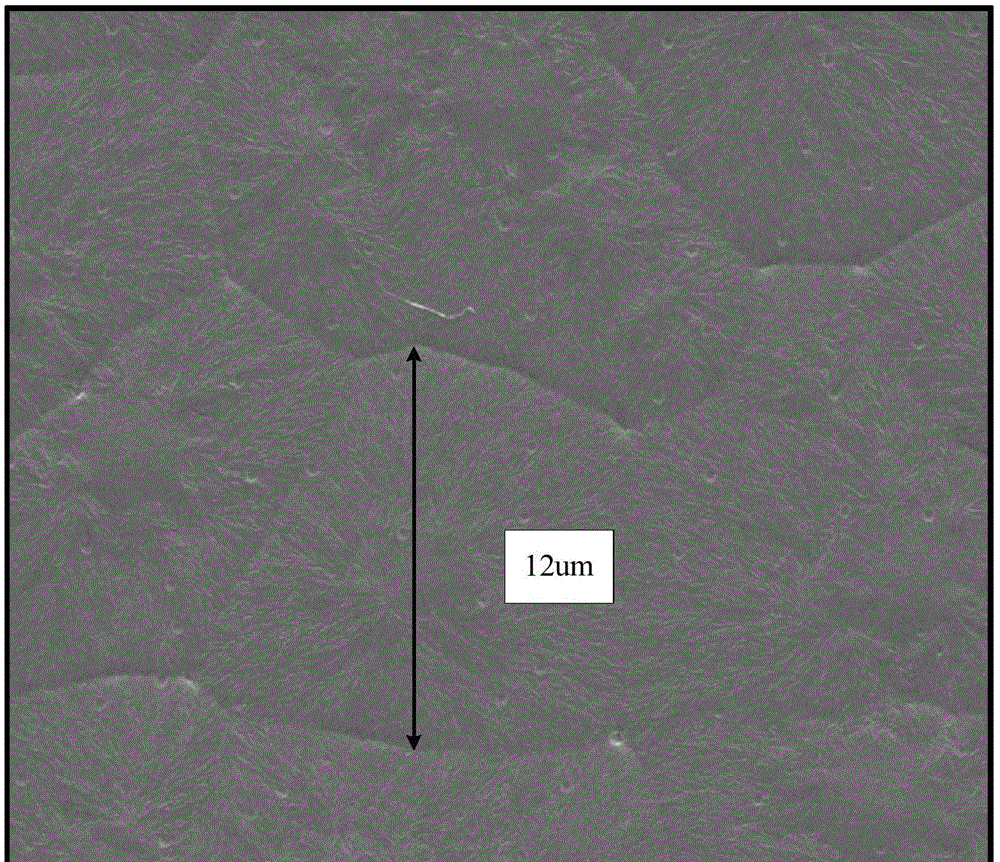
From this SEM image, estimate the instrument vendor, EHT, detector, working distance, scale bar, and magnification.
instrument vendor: FEI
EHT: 20 kV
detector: SE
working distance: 11 mm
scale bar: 10000 nm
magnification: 10 K X